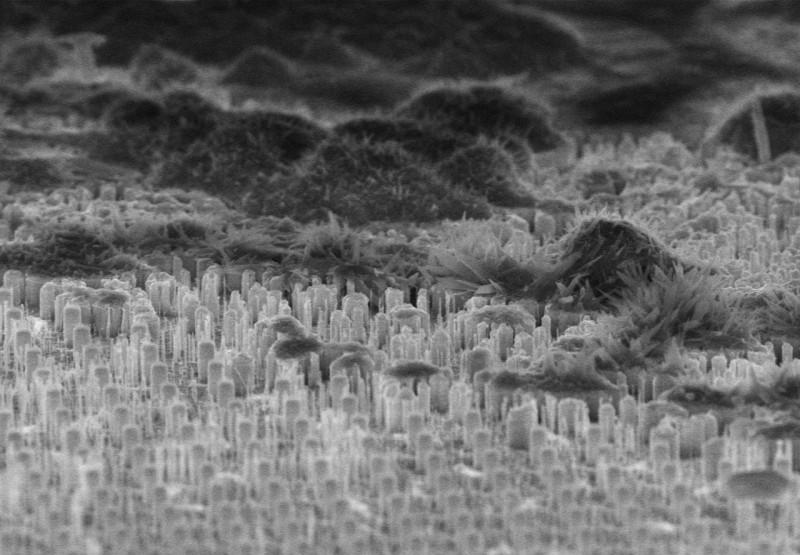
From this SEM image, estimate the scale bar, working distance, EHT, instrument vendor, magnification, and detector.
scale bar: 5000 nm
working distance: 8.2 mm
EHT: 10 kV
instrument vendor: Hitachi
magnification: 6 K X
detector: SE(U)